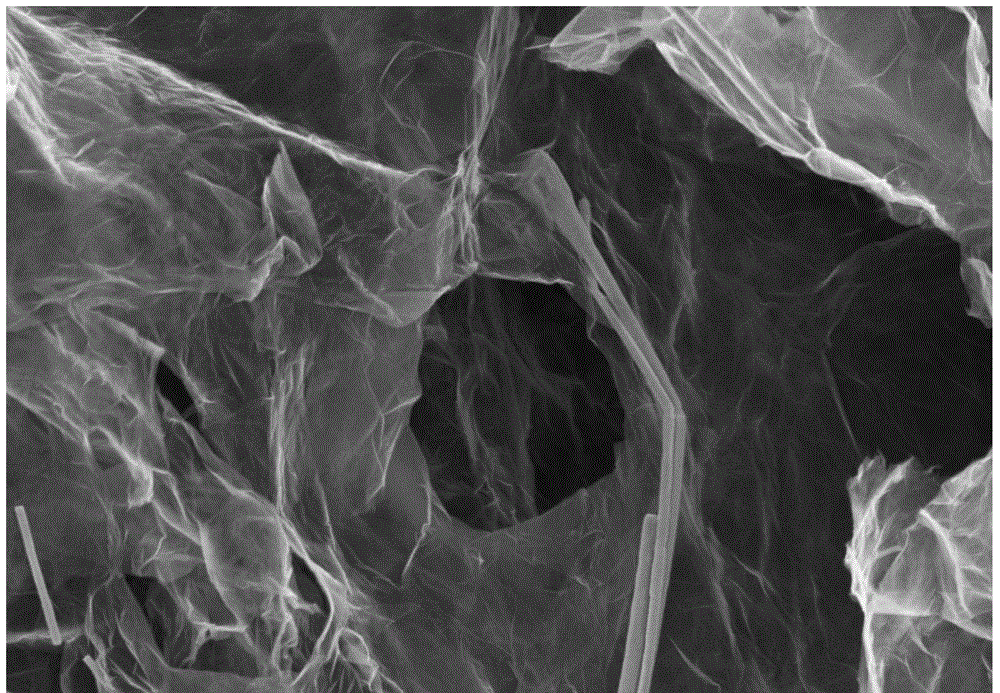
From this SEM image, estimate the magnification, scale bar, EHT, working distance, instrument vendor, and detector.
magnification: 20 K X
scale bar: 1000 nm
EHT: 3 kV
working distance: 8.9 mm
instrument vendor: Zeiss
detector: InLens